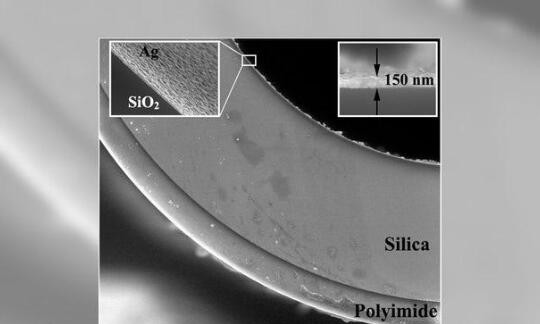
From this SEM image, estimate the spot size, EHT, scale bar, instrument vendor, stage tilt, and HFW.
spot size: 4.5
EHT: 20 kV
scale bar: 100000 nm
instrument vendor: FEI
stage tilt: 0°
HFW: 298 µm